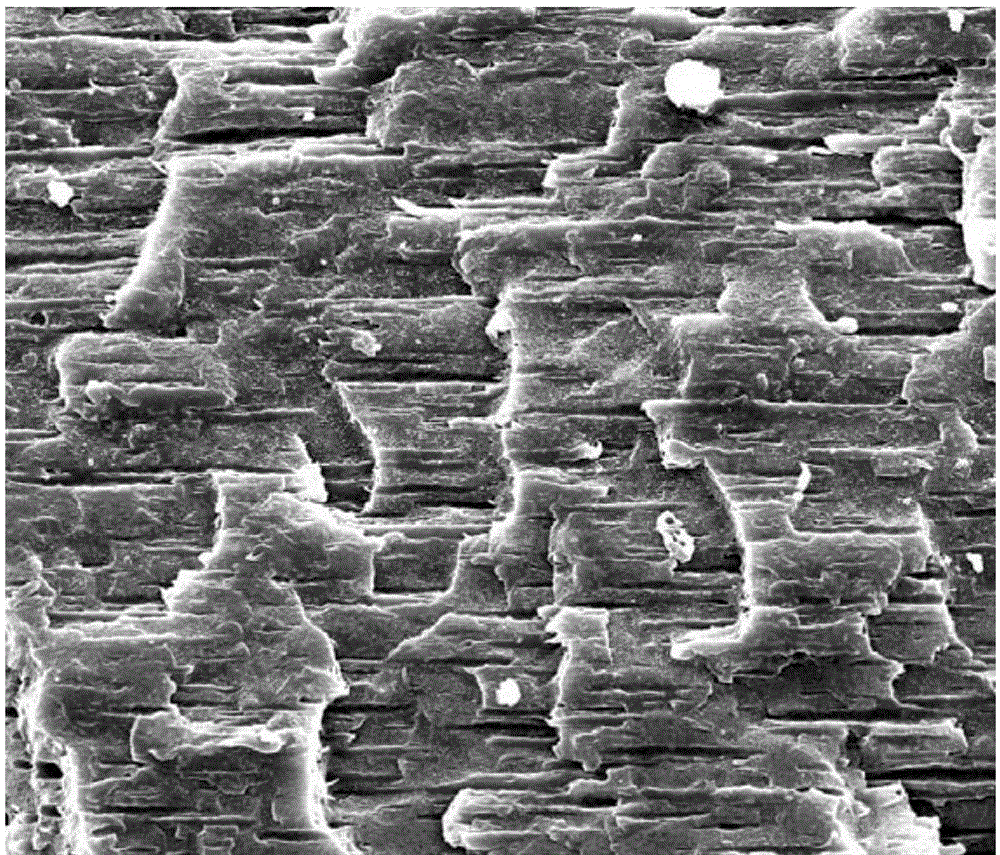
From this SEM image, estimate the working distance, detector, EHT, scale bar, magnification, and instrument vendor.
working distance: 12.7 mm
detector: SE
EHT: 20 kV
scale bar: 50000 nm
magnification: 2 K X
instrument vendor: FEI